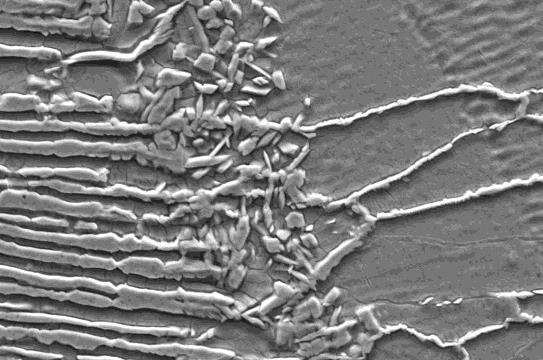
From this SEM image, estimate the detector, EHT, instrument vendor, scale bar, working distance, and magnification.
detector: SE1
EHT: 20 kV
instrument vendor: Zeiss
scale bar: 10000 nm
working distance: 10 mm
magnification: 3 K X